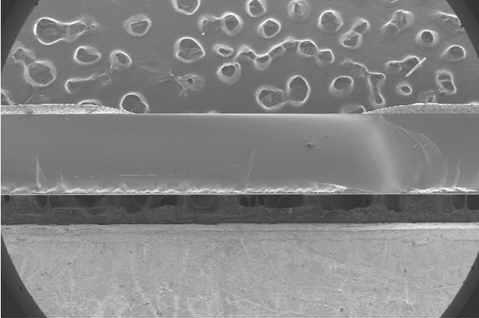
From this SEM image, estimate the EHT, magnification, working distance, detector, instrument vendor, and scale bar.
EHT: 5 kV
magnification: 0.03 K X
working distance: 10.1 mm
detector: SE(M)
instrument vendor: Hitachi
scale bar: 1000000 nm